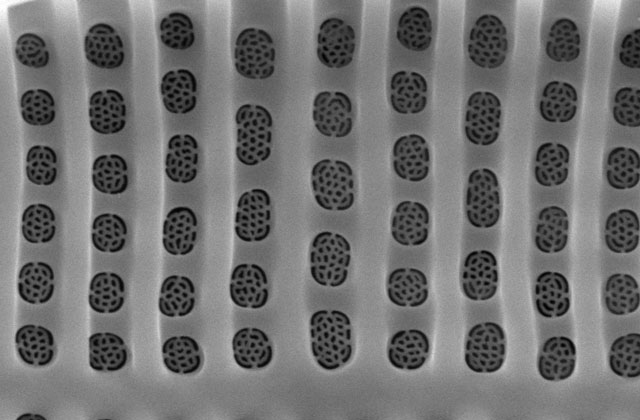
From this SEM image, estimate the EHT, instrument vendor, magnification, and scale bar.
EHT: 10 kV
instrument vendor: JEOL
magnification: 15 K X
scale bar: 1000 nm